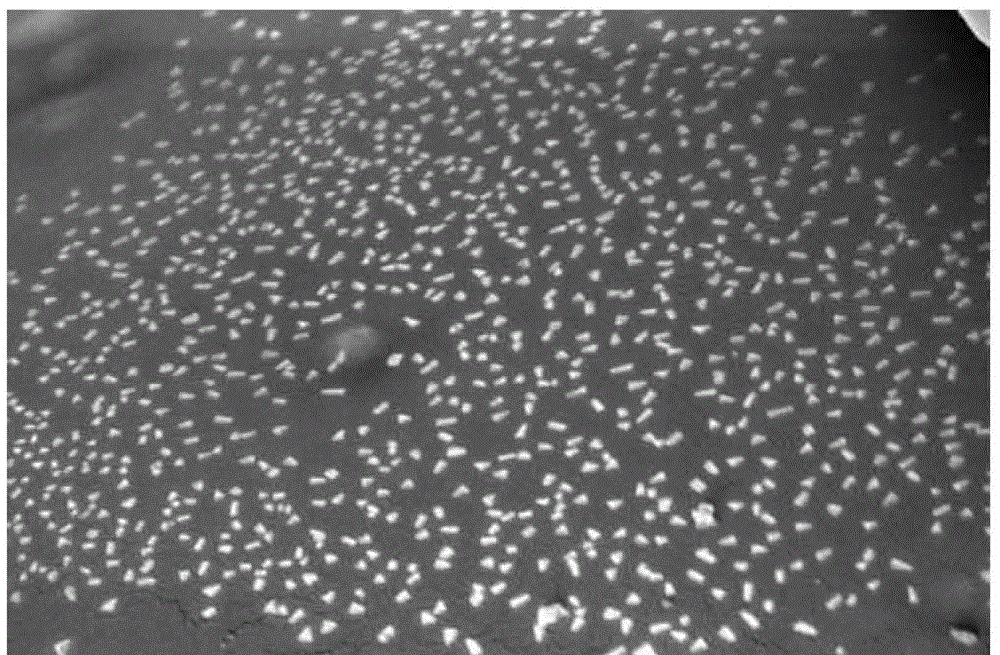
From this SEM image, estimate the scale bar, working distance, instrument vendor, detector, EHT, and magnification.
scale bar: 2000 nm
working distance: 13.1 mm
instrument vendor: Hitachi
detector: SE(M)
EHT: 5 kV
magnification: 25 K X